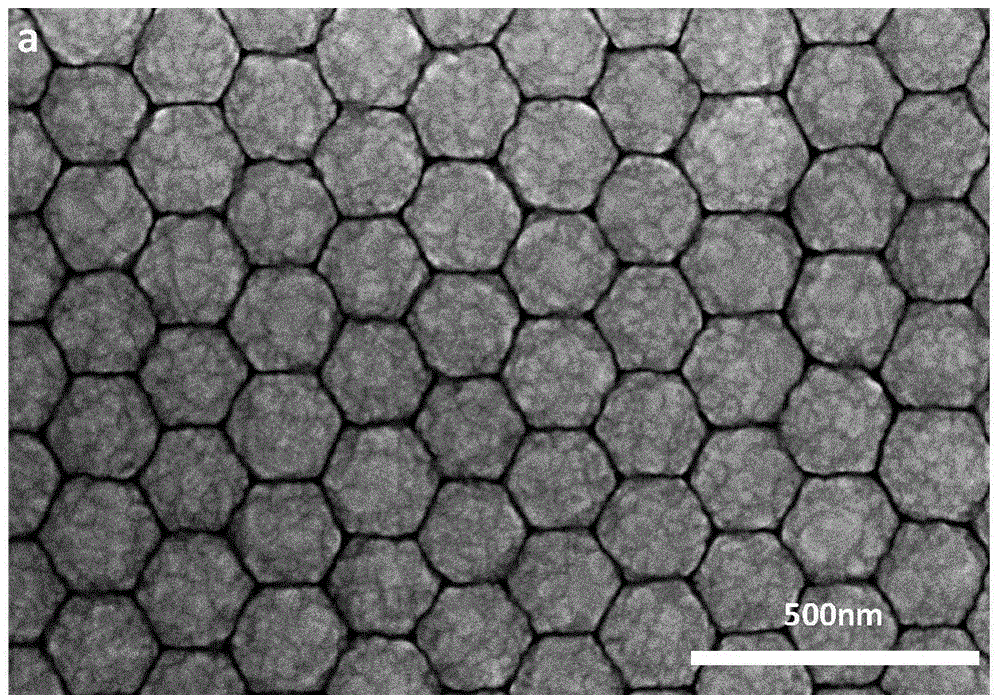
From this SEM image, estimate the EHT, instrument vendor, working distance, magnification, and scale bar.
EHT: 15 kV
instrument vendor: Hitachi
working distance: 8.5 mm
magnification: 70 K X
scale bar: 500 nm